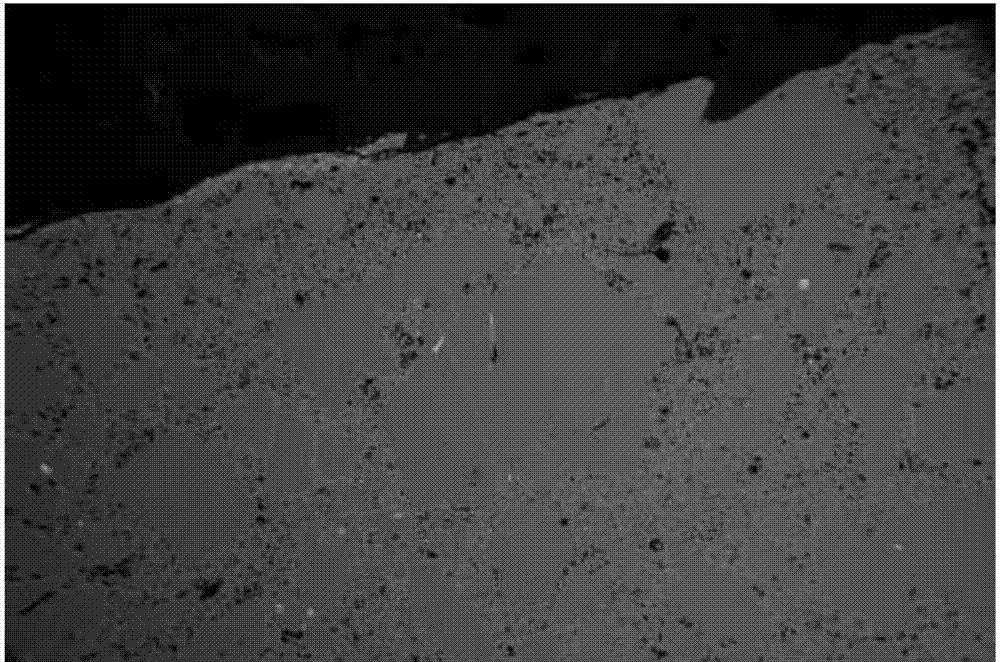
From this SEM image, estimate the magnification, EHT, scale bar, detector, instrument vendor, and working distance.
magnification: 0.019 K X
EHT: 20 kV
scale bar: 200000 nm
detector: CZ BSD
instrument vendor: Zeiss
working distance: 11 mm